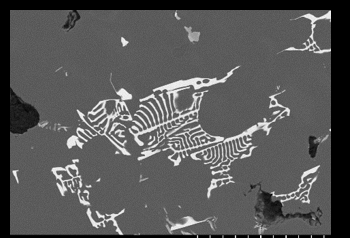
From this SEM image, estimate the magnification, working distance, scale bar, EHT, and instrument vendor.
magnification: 1 K X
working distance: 11.7 mm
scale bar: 50000 nm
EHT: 10 kV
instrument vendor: Hitachi